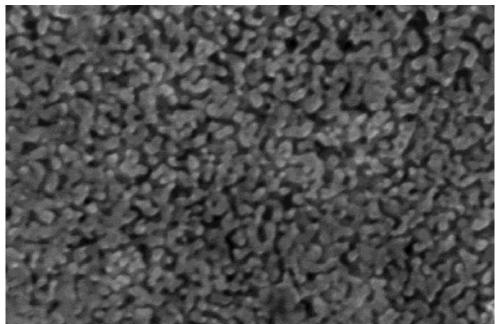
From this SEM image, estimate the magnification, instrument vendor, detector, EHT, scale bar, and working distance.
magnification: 100 K X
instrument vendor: Hitachi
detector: SE(M)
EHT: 15 kV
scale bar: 500 nm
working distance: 8.3 mm